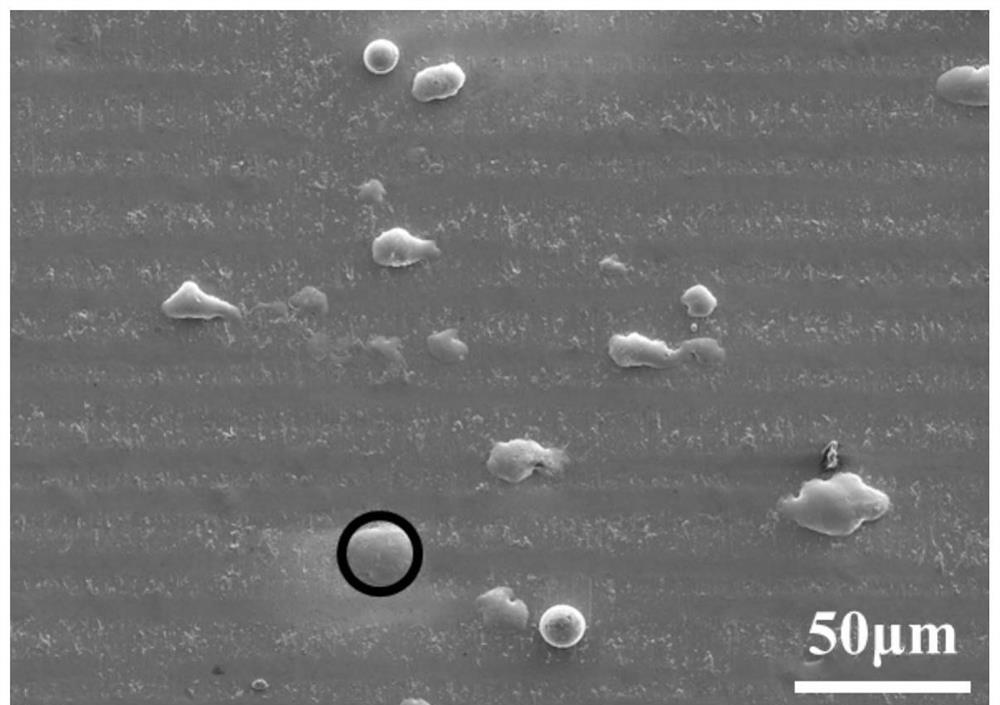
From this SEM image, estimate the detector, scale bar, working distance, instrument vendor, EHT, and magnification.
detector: SE2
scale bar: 30000 nm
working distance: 13.6 mm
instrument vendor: Zeiss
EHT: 10 kV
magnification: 0.2 K X